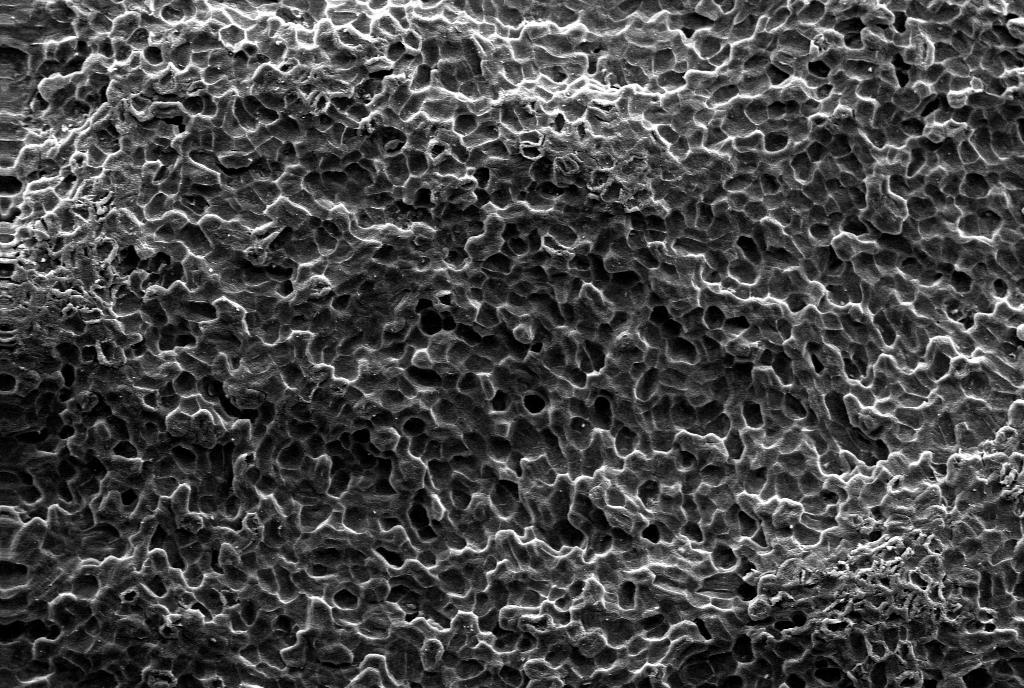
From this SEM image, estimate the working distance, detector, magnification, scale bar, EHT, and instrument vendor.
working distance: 8 mm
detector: SE1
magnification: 0.1 K X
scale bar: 100000 nm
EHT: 20 kV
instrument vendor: Zeiss